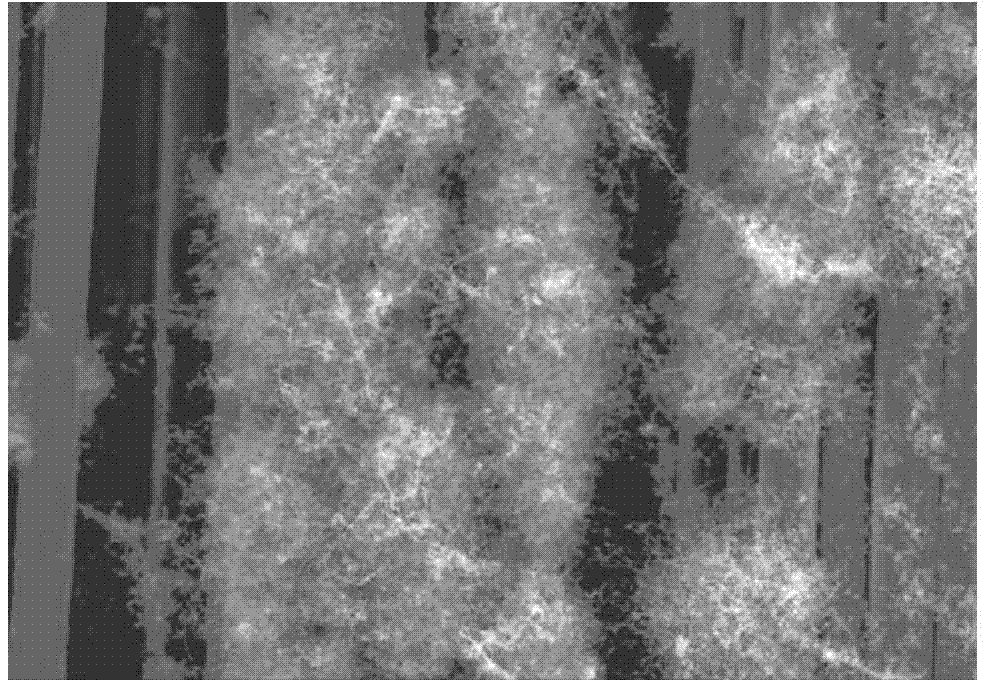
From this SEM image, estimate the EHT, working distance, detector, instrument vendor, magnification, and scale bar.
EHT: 15 kV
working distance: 11.8 mm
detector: SE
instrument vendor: Hitachi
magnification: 1 K X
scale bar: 50000 nm